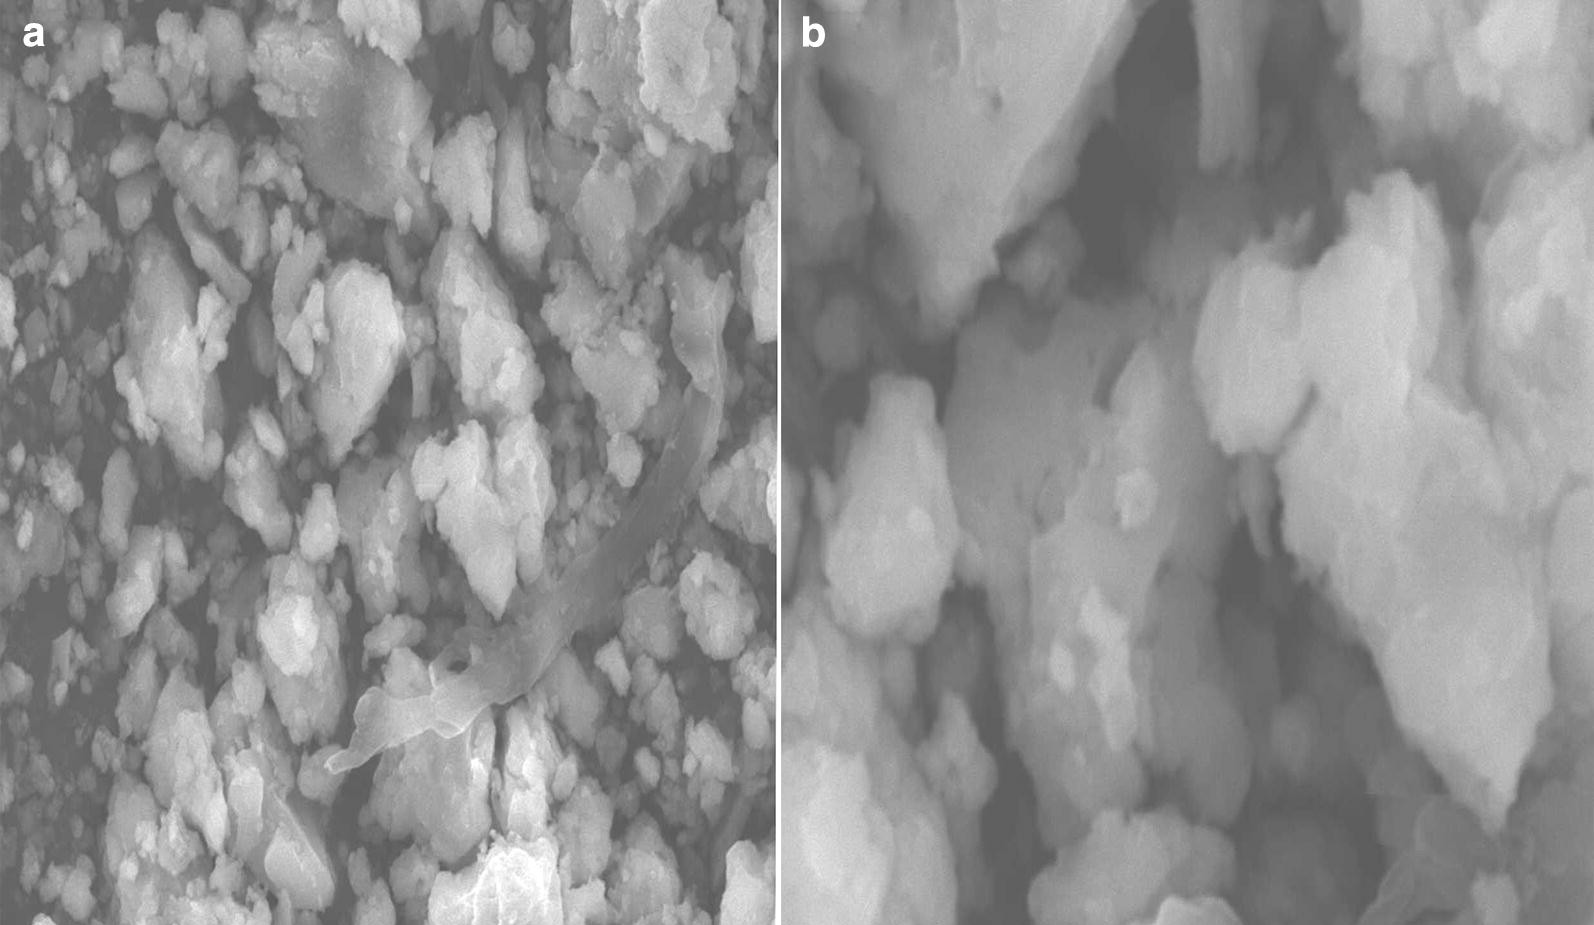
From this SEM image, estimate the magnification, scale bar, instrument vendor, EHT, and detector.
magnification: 3 K X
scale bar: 5000 nm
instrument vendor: JEOL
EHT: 20 kV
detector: SEI